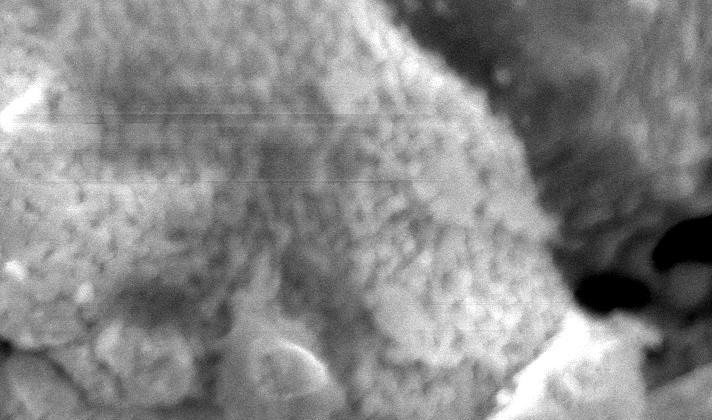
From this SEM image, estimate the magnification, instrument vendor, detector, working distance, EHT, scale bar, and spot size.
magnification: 5 K X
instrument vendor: FEI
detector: SE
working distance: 9.8 mm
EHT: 25 kV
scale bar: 5000 nm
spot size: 3